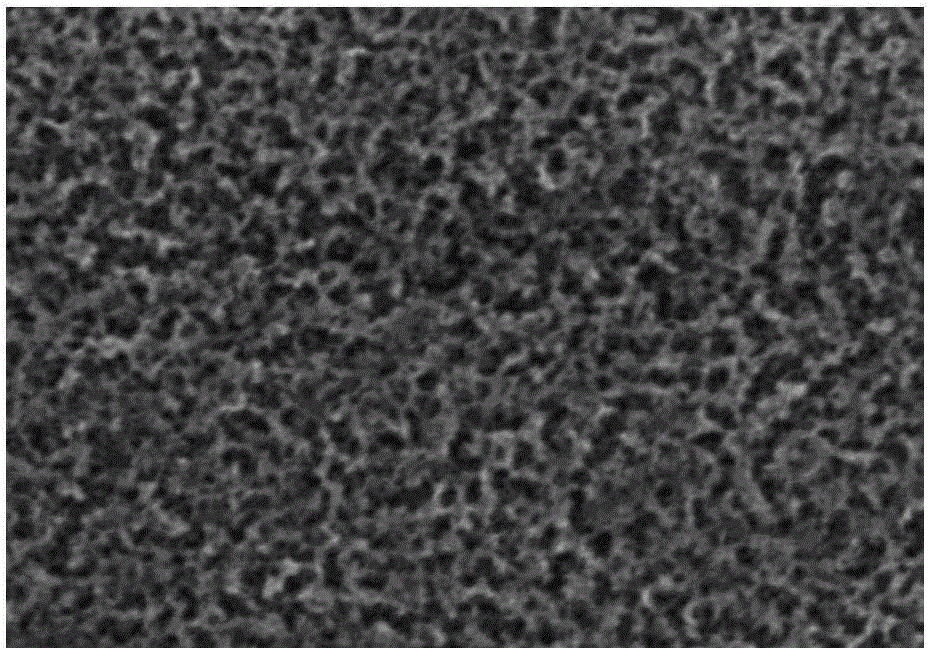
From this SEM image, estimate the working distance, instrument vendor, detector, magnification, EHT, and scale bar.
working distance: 13.8 mm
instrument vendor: Hitachi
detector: SE(M)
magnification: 50 K X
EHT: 15 kV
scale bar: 200 nm